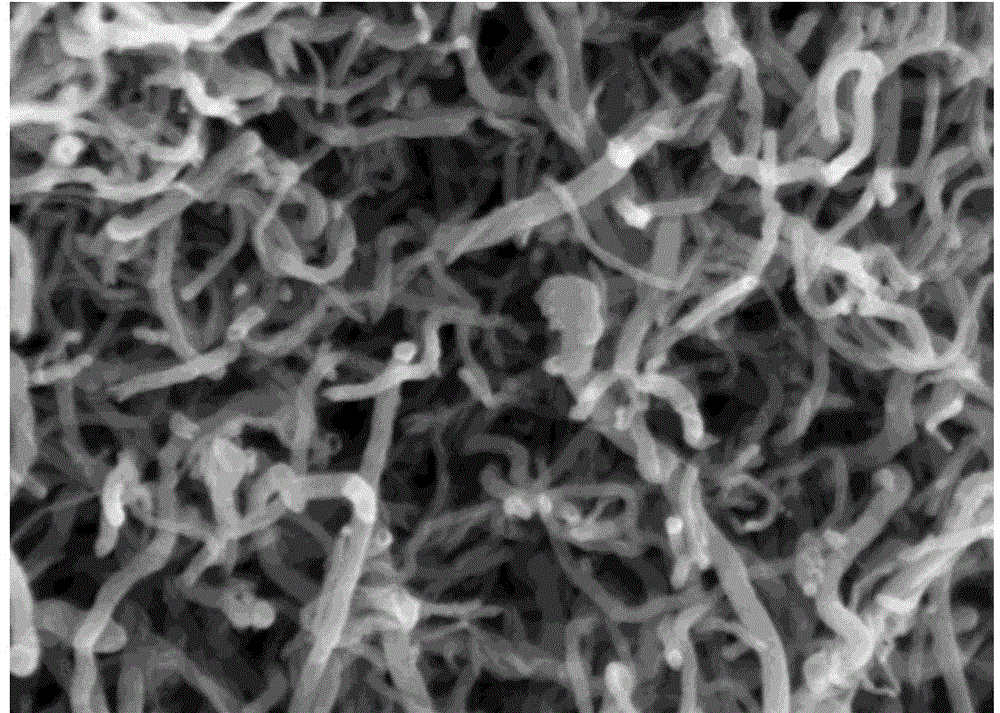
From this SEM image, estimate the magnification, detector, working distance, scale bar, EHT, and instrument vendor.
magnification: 60 K X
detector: SE(M)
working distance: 7.9 mm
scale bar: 500 nm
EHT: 3 kV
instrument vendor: Hitachi